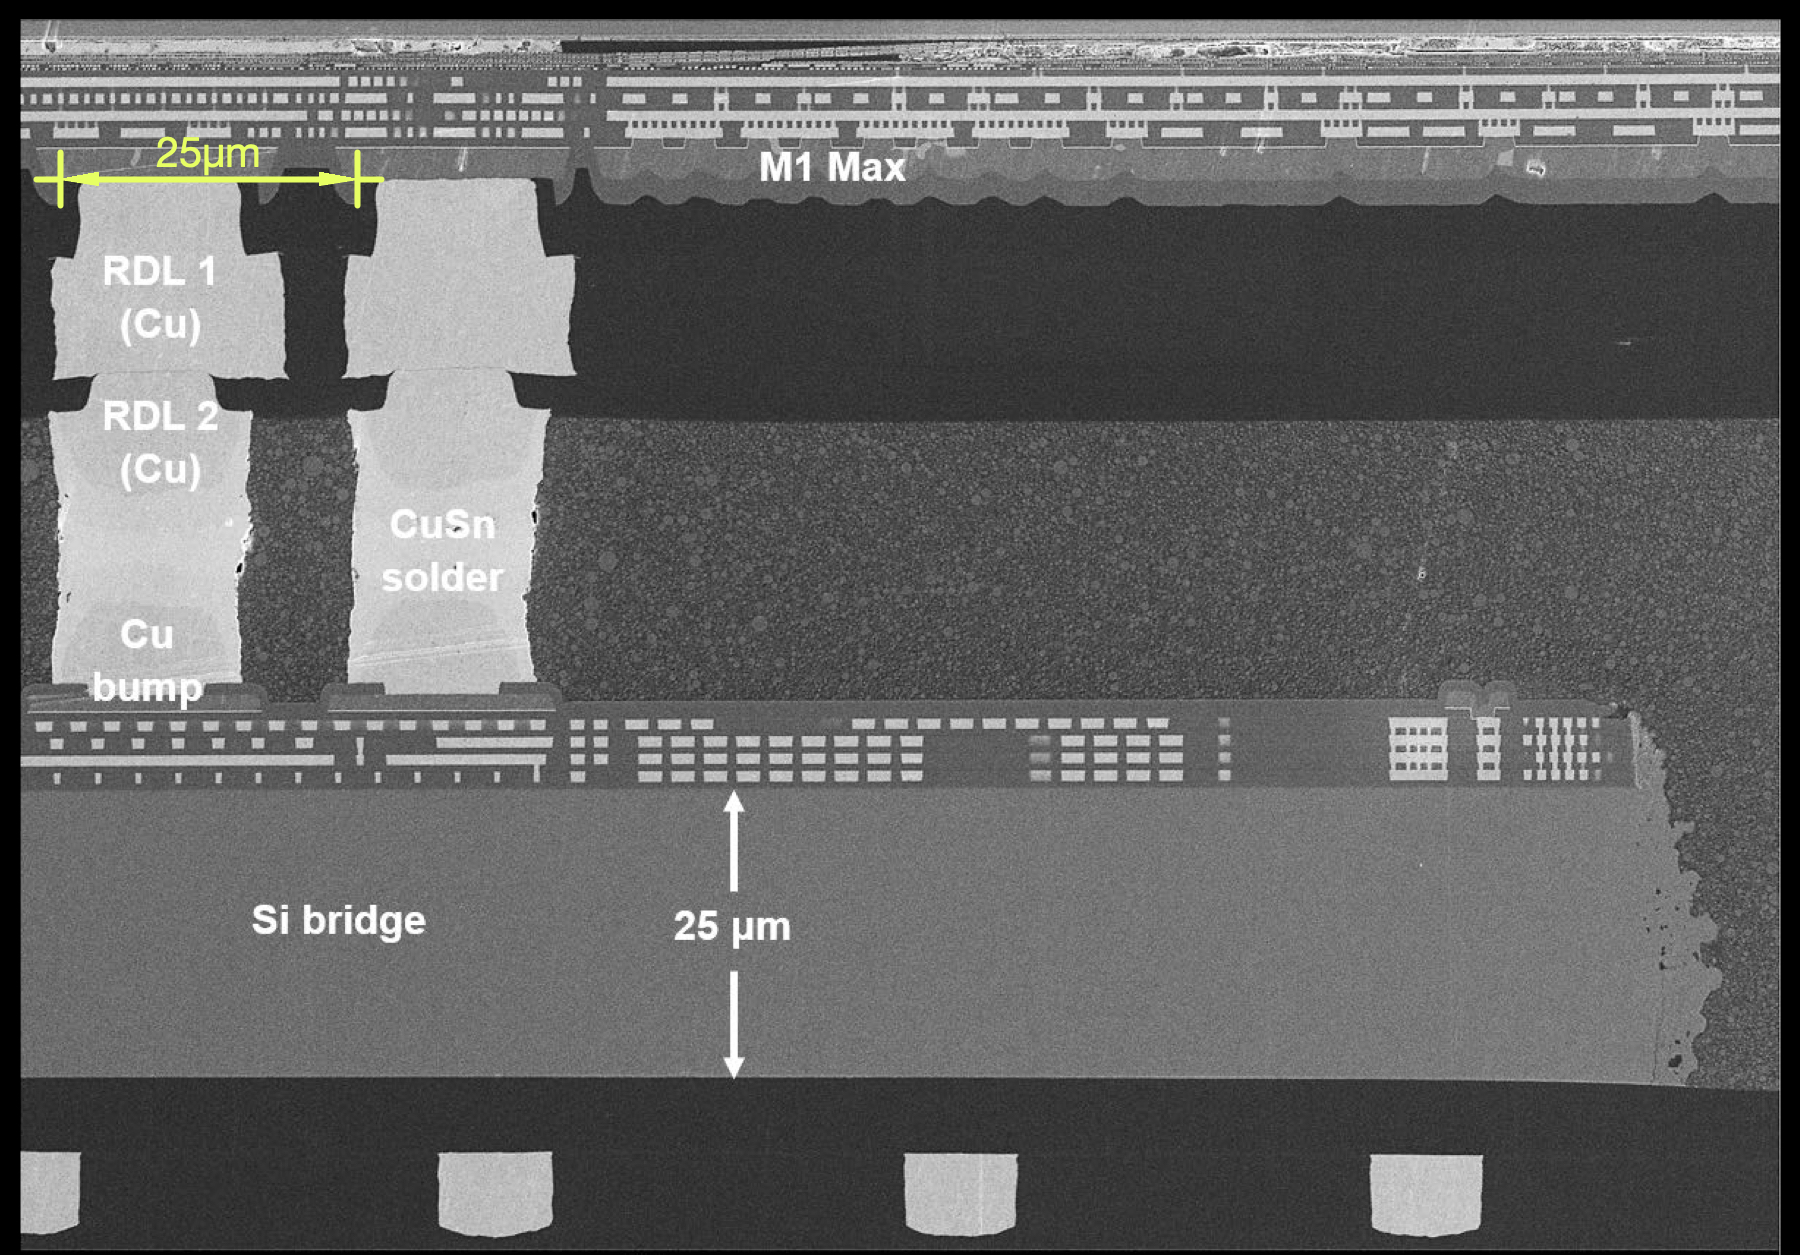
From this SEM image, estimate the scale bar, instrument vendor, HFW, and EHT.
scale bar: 20000 nm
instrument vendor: Zeiss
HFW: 151.1 µm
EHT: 5 kV